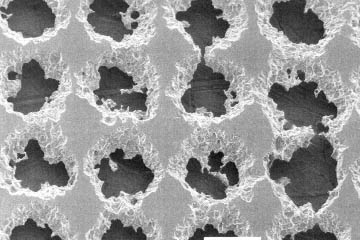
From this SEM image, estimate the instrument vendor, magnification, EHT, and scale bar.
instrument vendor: JEOL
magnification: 0.2 K X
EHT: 20 kV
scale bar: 100000 nm